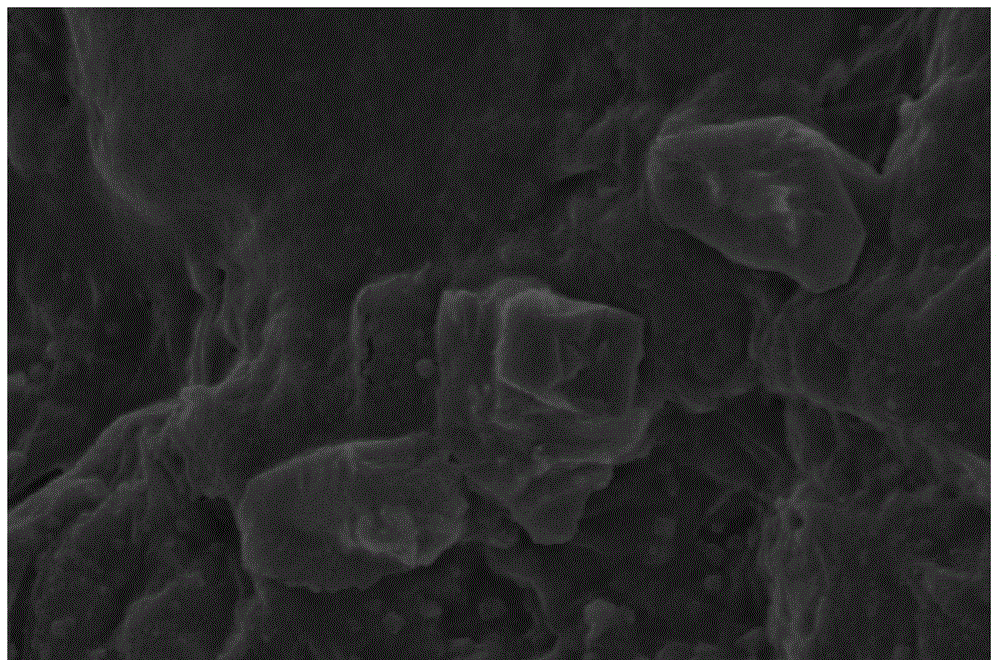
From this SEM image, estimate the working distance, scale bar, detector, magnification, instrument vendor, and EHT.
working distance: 17.8 mm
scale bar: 10000 nm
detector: SE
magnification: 3 K X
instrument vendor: Hitachi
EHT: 10 kV